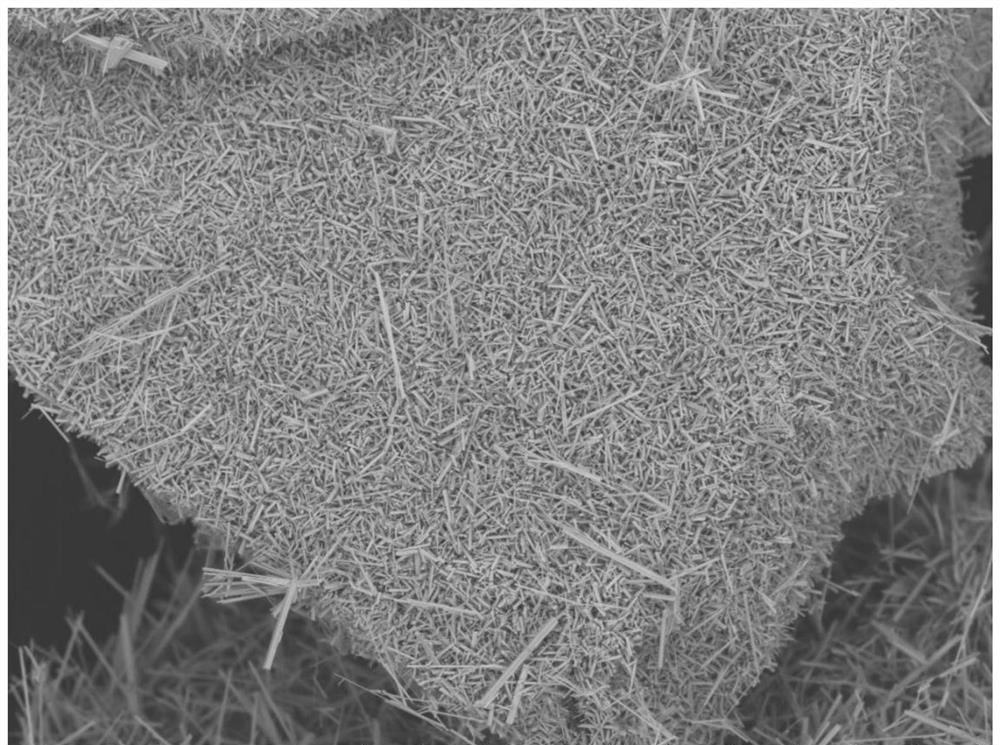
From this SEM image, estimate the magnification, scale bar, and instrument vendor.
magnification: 0.5 K X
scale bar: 200000 nm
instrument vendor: Hitachi